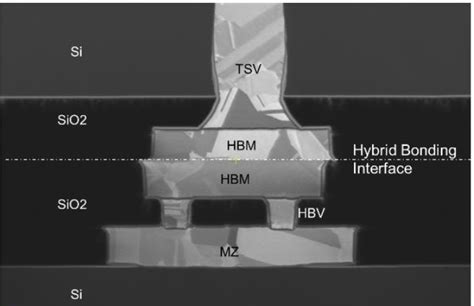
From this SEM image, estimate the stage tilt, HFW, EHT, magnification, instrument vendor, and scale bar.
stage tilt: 0°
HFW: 6.38 µm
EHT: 30 kV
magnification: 65 K X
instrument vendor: FEI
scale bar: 2000 nm